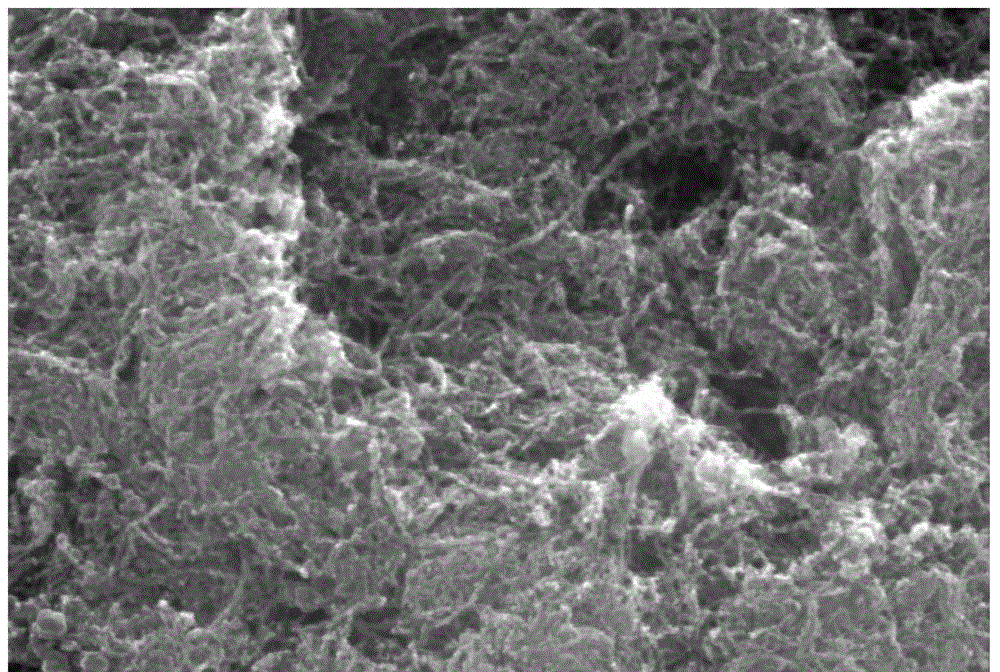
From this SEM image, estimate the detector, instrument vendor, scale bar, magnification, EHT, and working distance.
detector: ETD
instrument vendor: FEI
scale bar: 1000 nm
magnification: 100 K X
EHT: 20 kV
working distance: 10.1 mm